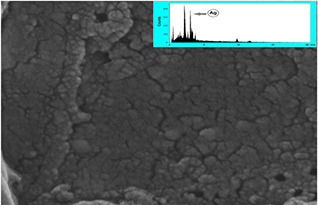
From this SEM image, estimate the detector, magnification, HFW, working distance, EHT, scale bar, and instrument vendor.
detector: SE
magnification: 187 K X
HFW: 1.11 µm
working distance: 5.47 mm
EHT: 25 kV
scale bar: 200 nm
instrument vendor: Tescan